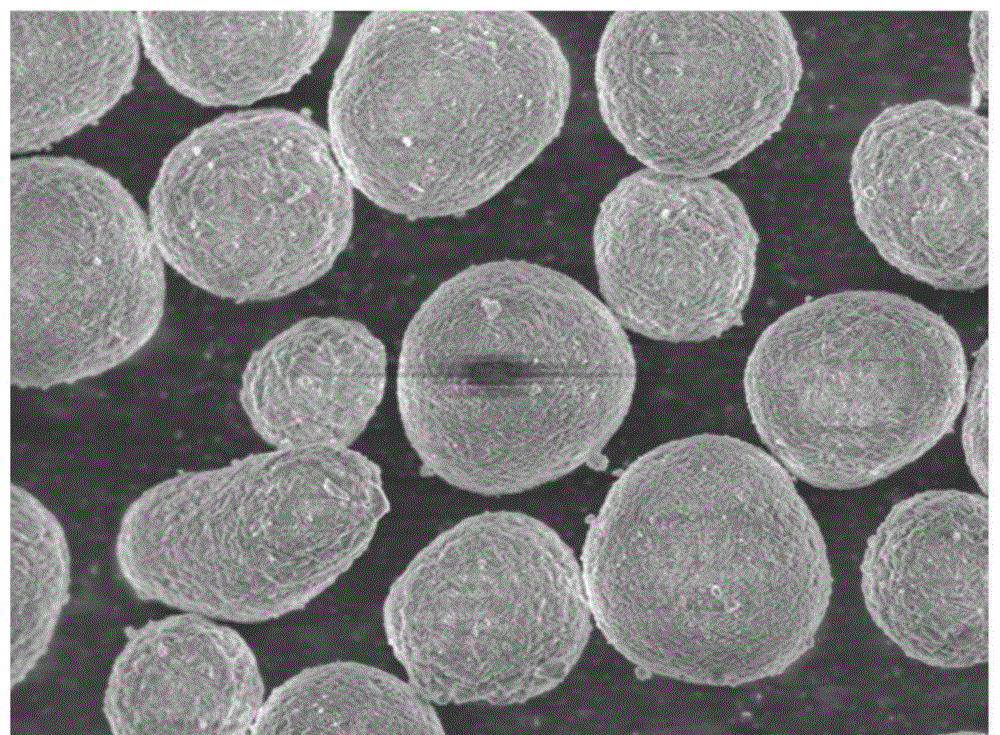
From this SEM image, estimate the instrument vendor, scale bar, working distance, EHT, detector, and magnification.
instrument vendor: JEOL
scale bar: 1000 nm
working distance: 7.8 mm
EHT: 5 kV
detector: SEI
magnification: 3 K X